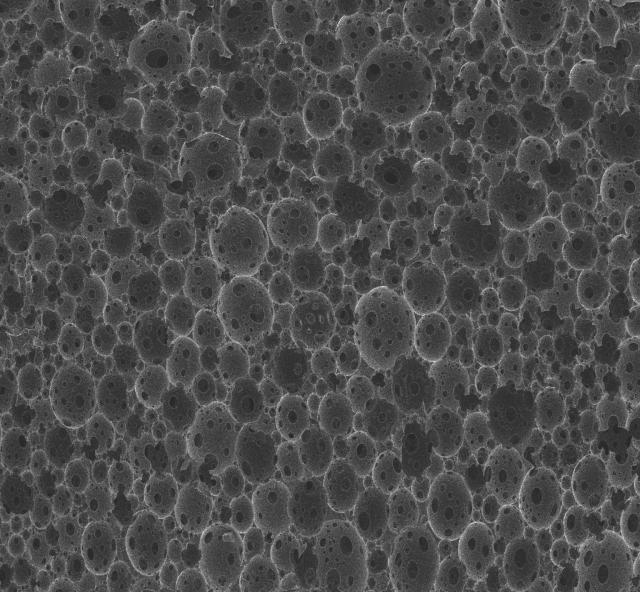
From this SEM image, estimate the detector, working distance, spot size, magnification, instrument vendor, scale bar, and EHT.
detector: ETD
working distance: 9 mm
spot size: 3.5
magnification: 0.5 K X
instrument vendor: FEI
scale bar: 300000 nm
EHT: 7 kV